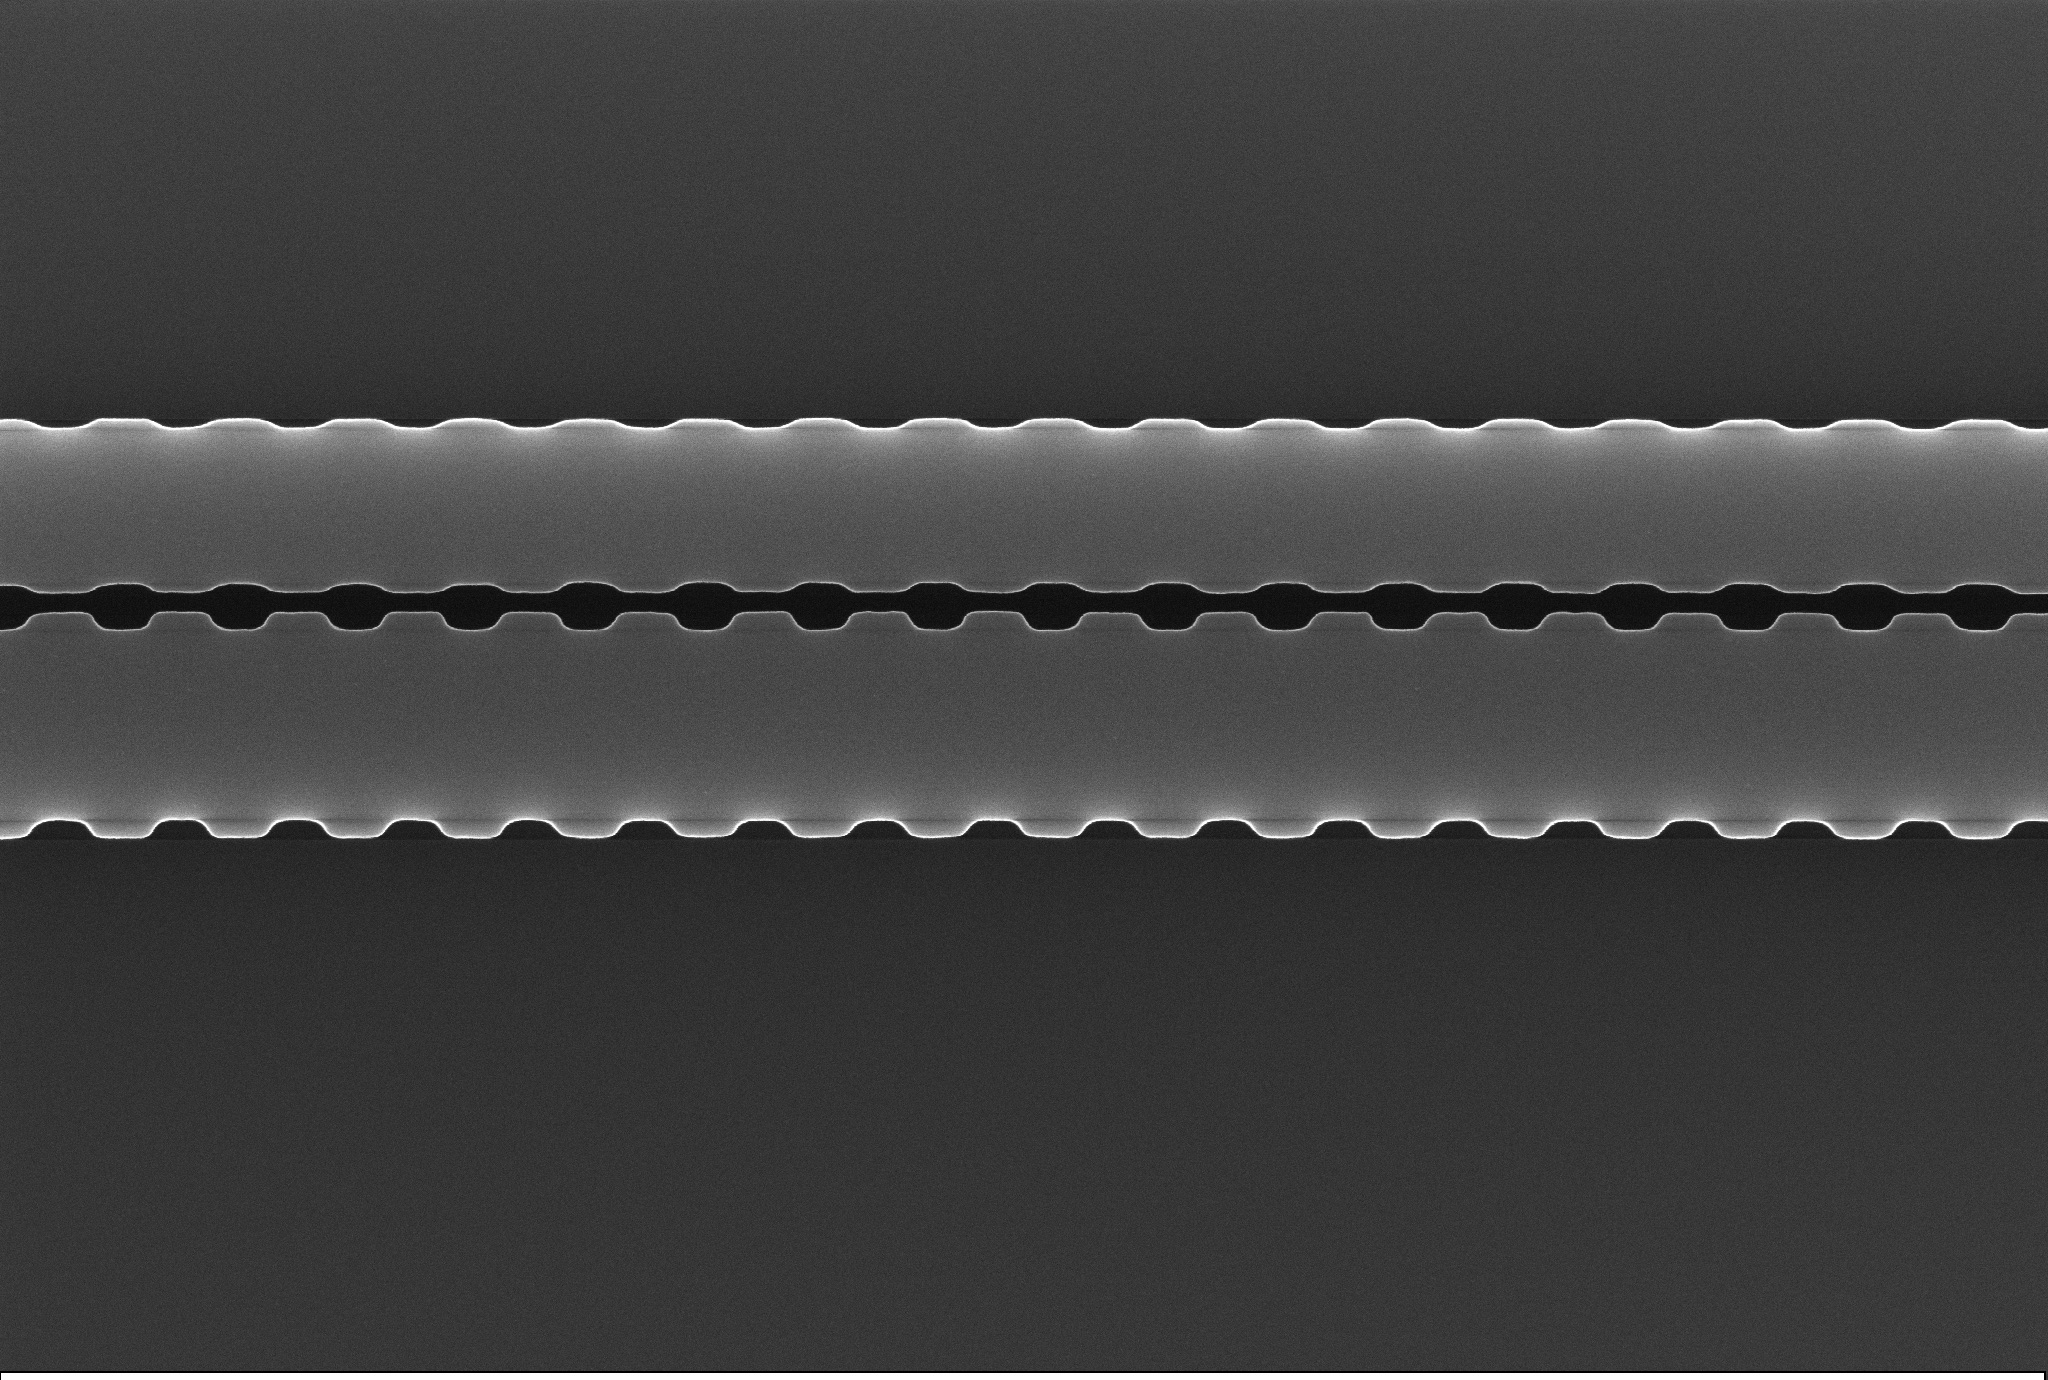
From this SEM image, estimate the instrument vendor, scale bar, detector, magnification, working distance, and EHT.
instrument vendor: Zeiss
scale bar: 200 nm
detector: InLens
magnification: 20.41 K X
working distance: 6.7 mm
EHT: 15 kV